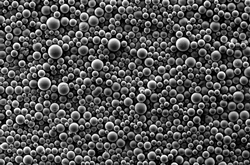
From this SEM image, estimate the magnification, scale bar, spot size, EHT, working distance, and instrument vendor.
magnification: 0.196 K X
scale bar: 100000 nm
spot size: -1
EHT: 5 kV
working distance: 8 mm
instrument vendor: other